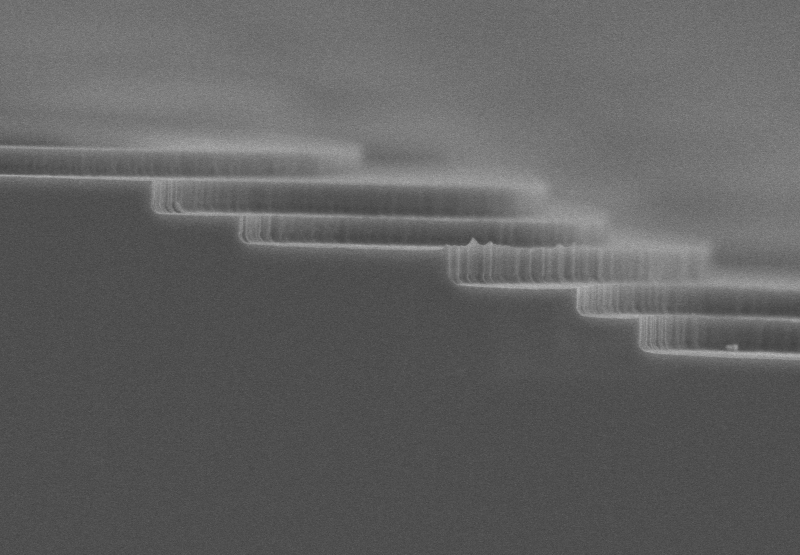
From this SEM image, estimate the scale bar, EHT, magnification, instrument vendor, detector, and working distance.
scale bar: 2000 nm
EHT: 3 kV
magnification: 20 K X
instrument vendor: Hitachi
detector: SE(UL)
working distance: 10 mm